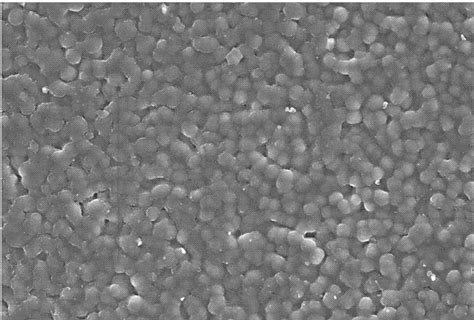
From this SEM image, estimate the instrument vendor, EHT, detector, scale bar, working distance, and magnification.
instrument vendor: Hitachi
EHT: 15 kV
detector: SE(UL)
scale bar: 50000 nm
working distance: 16.1 mm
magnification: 1 K X